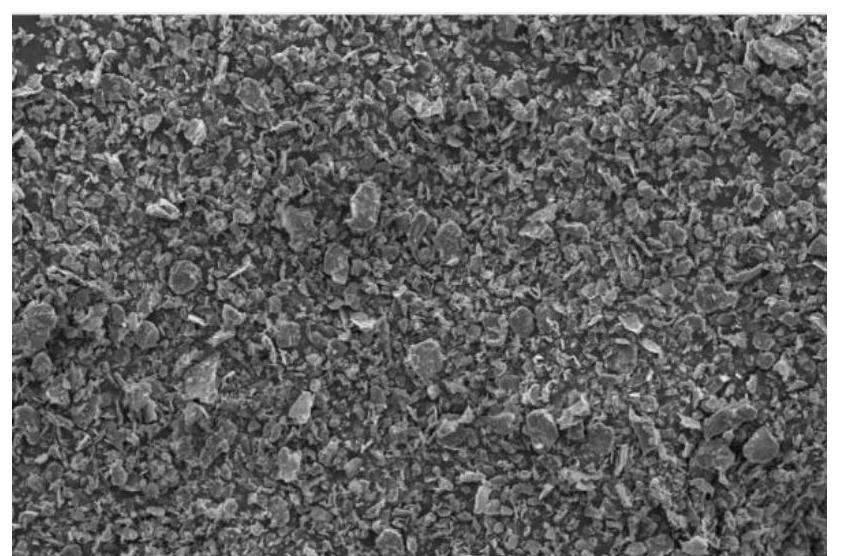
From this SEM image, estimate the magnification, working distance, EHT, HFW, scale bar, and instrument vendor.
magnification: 1 K X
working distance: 10.2 mm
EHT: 10 kV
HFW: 414 µm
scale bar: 100000 nm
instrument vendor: FEI